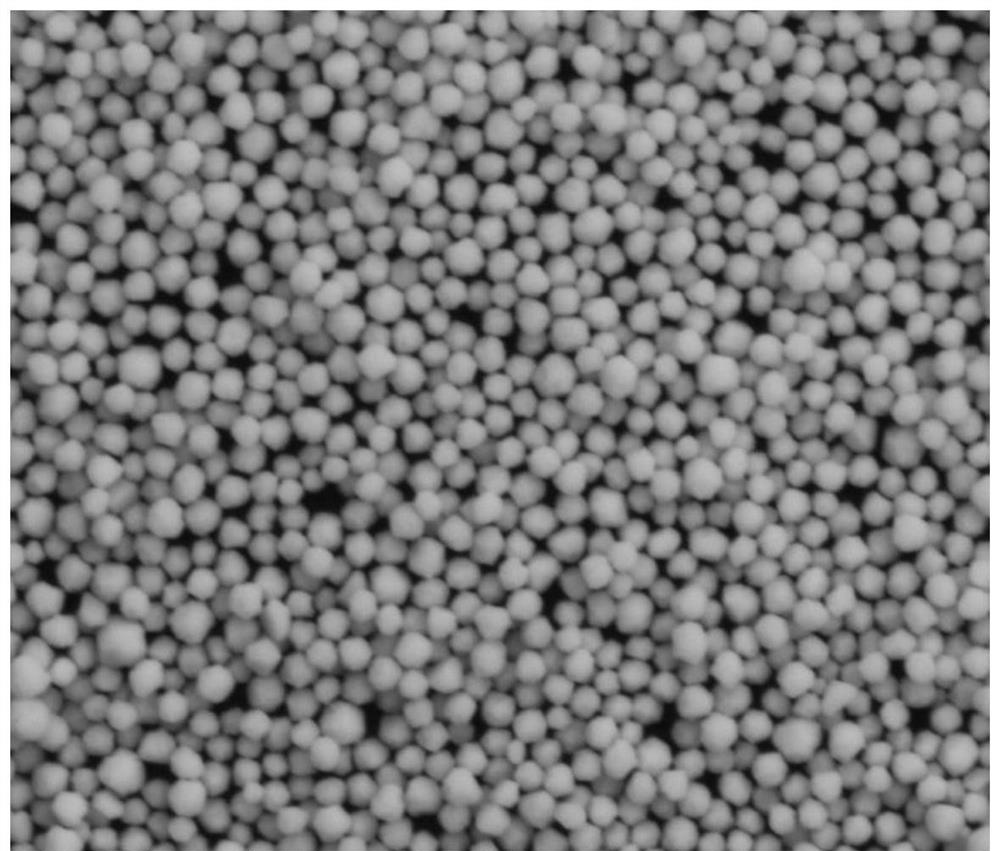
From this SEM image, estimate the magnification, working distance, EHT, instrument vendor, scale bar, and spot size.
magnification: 10 K X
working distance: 9.6 mm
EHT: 30 kV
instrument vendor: FEI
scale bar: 10000 nm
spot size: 4.5